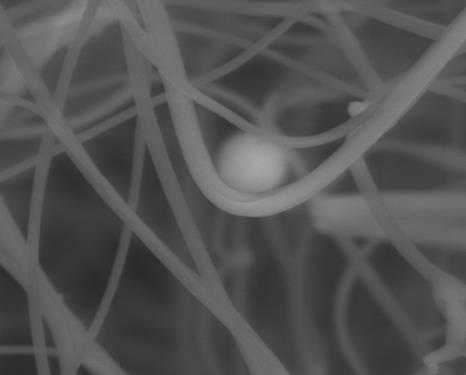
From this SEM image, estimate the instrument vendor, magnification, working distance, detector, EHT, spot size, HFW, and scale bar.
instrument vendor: FEI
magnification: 10 K X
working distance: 5.4 mm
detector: LVD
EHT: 18 kV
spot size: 4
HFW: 19.9 µm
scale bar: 5000 nm